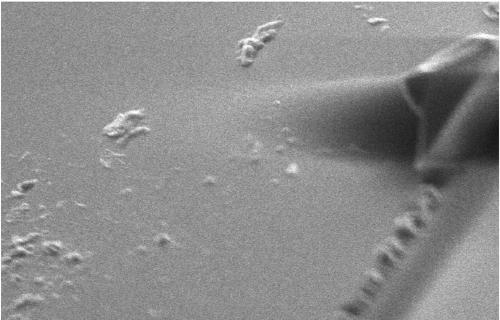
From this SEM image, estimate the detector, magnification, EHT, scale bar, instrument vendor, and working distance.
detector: SE1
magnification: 4.18 K X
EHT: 5 kV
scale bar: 2000 nm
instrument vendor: Zeiss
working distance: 5.5 mm